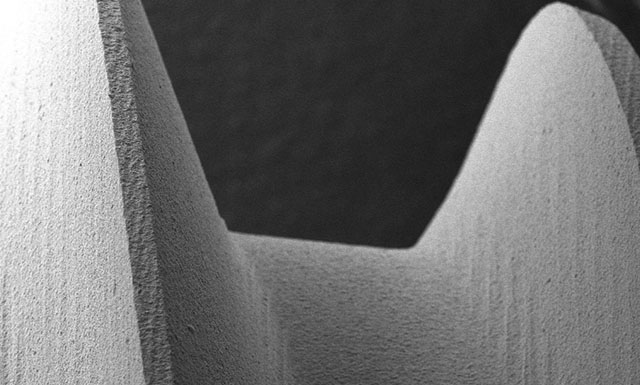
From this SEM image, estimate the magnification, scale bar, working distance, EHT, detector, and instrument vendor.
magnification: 0.2 K X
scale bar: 200000 nm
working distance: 13.5 mm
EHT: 20 kV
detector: SE1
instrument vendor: Zeiss